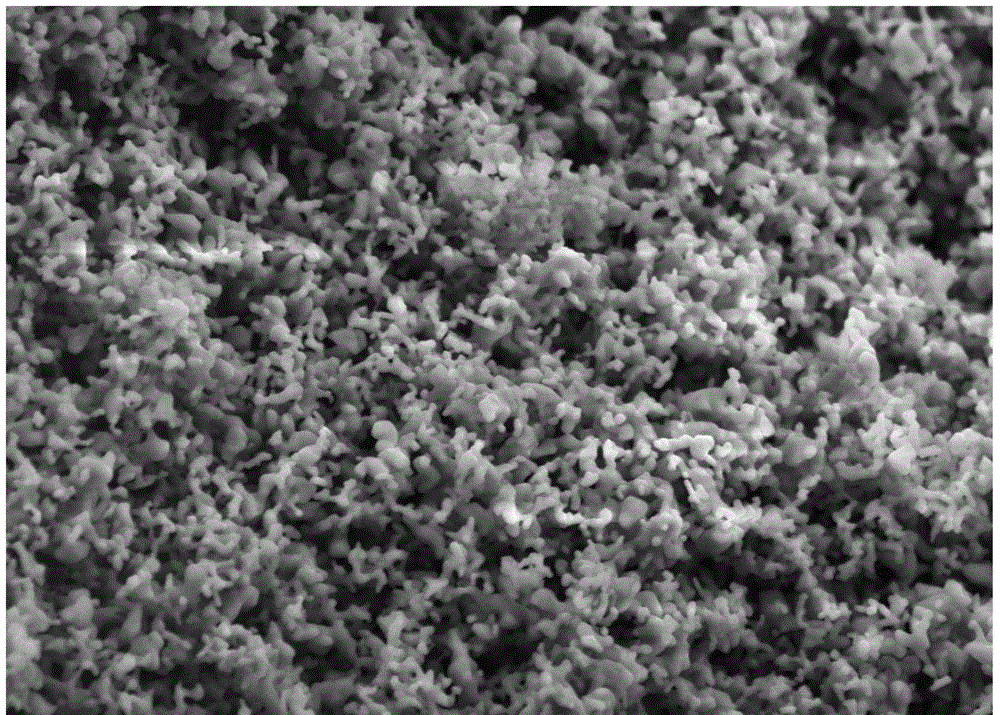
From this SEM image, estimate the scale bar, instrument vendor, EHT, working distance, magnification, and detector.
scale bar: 1000 nm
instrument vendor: Zeiss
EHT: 5 kV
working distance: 5.3 mm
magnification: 10 K X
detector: SE2